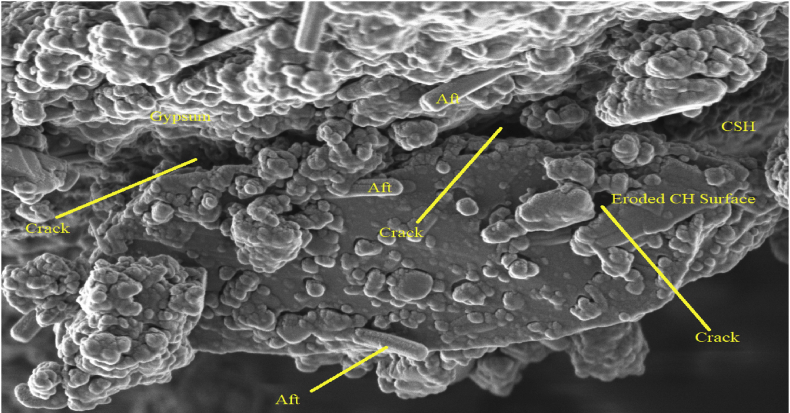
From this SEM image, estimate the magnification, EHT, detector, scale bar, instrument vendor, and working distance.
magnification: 63.19 K X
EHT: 1 kV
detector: InLens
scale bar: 200 nm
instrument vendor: Zeiss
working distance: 2.7 mm